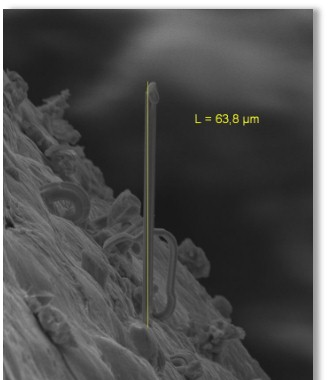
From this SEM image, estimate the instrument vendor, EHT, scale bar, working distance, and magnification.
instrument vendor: Zeiss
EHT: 20 kV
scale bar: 20000 nm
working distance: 31 mm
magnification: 1.2 K X